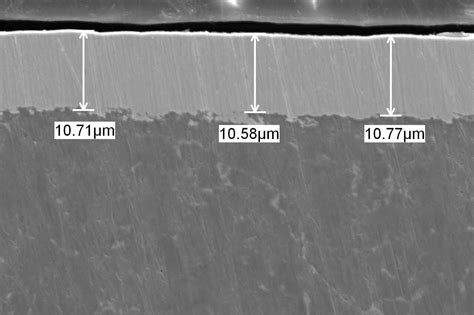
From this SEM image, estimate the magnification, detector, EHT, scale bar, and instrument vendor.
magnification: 2 K X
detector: SEI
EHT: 15 kV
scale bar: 10000 nm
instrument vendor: JEOL